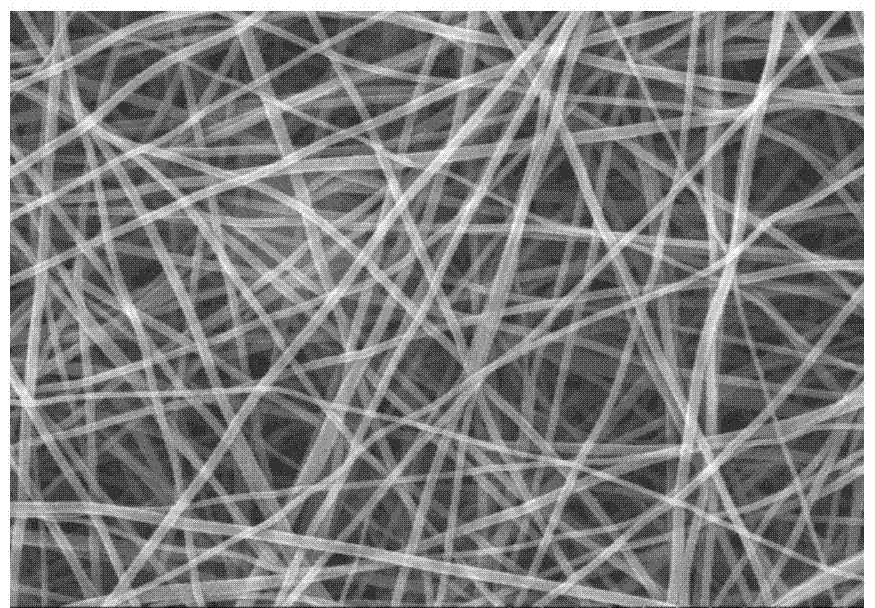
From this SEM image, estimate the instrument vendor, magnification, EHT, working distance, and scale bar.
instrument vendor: Hitachi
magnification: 5 K X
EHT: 20 kV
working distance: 12.4 mm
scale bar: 10000 nm